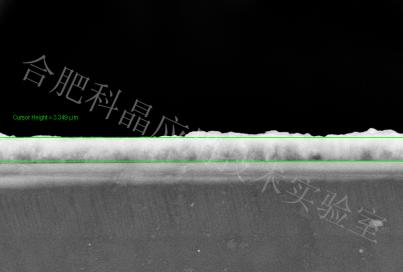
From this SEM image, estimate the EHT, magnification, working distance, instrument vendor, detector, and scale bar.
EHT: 20 kV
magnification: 2 K X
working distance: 15.6 mm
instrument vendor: Zeiss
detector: SE2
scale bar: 2000 nm